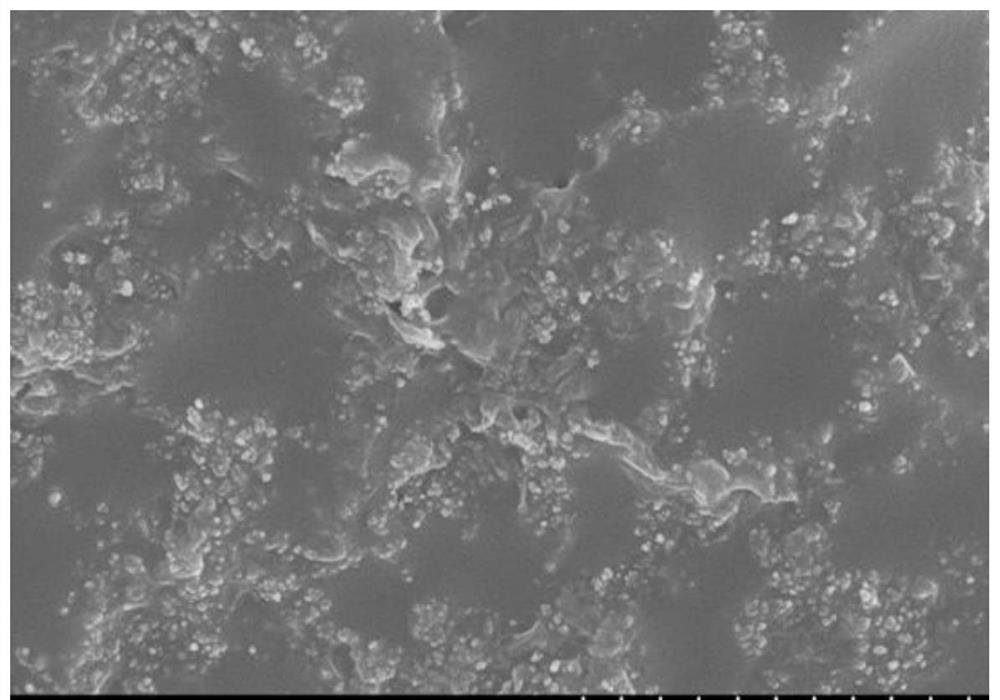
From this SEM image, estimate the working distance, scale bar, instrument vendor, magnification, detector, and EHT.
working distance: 8.6 mm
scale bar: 2000 nm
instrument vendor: Hitachi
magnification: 25 K X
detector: SE(M)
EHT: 5 kV